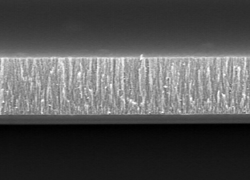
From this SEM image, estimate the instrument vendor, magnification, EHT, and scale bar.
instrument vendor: JEOL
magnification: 10 K X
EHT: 3 kV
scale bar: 2000 nm